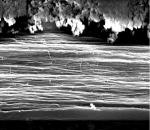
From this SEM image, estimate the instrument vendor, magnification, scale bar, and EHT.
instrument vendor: JEOL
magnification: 5.5 K X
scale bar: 2000 nm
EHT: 15 kV